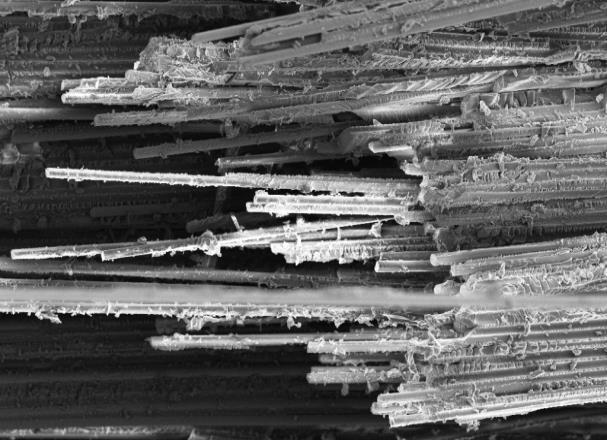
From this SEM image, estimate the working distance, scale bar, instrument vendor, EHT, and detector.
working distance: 9.8 mm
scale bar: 100000 nm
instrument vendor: Zeiss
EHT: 7 kV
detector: SE2